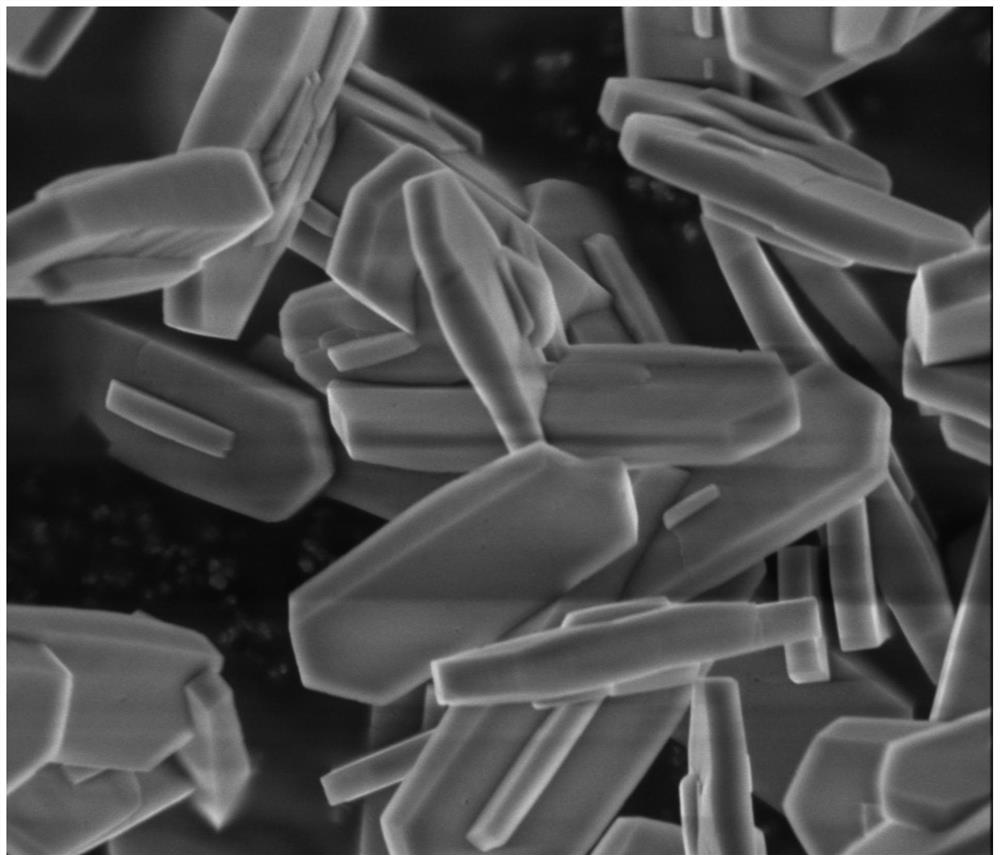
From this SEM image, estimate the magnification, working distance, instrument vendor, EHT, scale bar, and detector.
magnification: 50 K X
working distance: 4.9 mm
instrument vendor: FEI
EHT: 3 kV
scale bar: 1000 nm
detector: SE